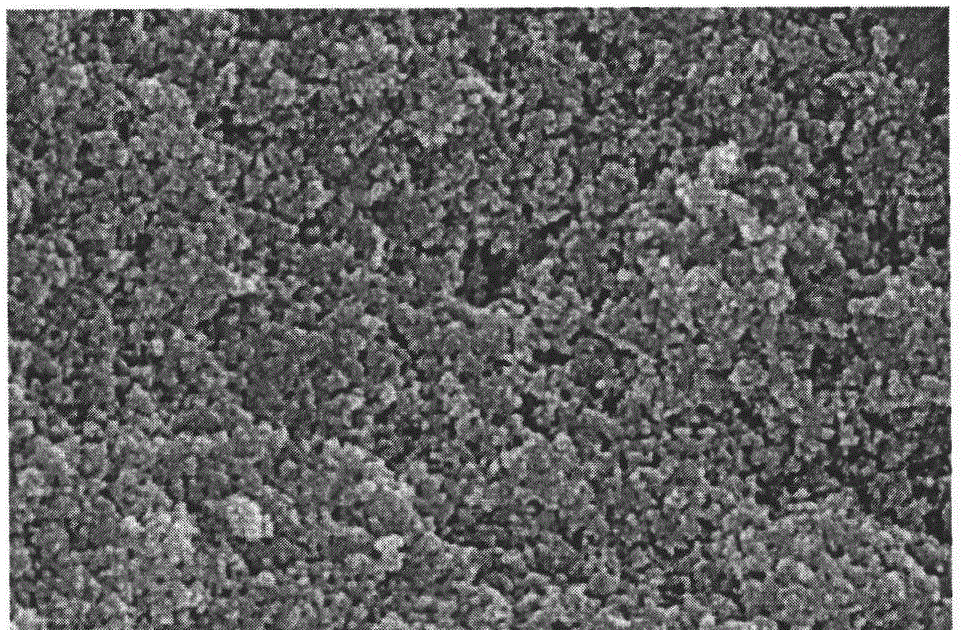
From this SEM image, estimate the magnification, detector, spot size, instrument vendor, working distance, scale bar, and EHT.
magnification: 150 K X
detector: TLD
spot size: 3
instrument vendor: FEI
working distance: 6.7 mm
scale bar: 500 nm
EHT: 5 kV